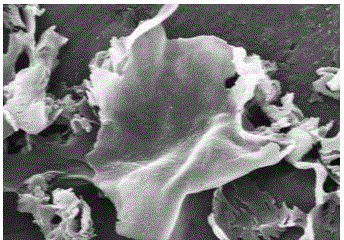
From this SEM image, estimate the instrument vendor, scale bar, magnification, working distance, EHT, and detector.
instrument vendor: Hitachi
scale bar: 1000 nm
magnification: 50 K X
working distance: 8.5 mm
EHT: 5 kV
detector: SE(U)